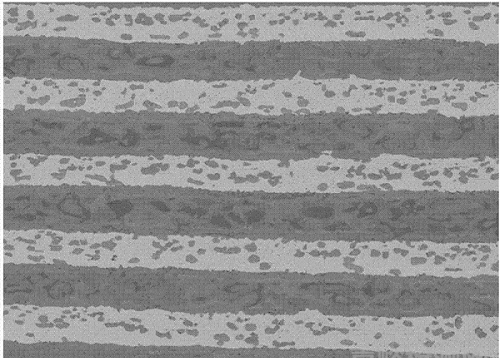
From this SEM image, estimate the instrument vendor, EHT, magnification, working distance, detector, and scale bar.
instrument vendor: JEOL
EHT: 15 kV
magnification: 0.5 K X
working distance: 11 mm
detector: COMPO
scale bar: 10000 nm